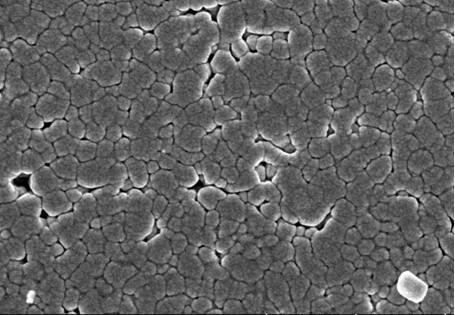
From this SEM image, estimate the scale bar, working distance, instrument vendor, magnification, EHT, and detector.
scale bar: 1000 nm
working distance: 8.2 mm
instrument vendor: Hitachi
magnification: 50 K X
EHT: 3 kV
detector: SE(TUL)